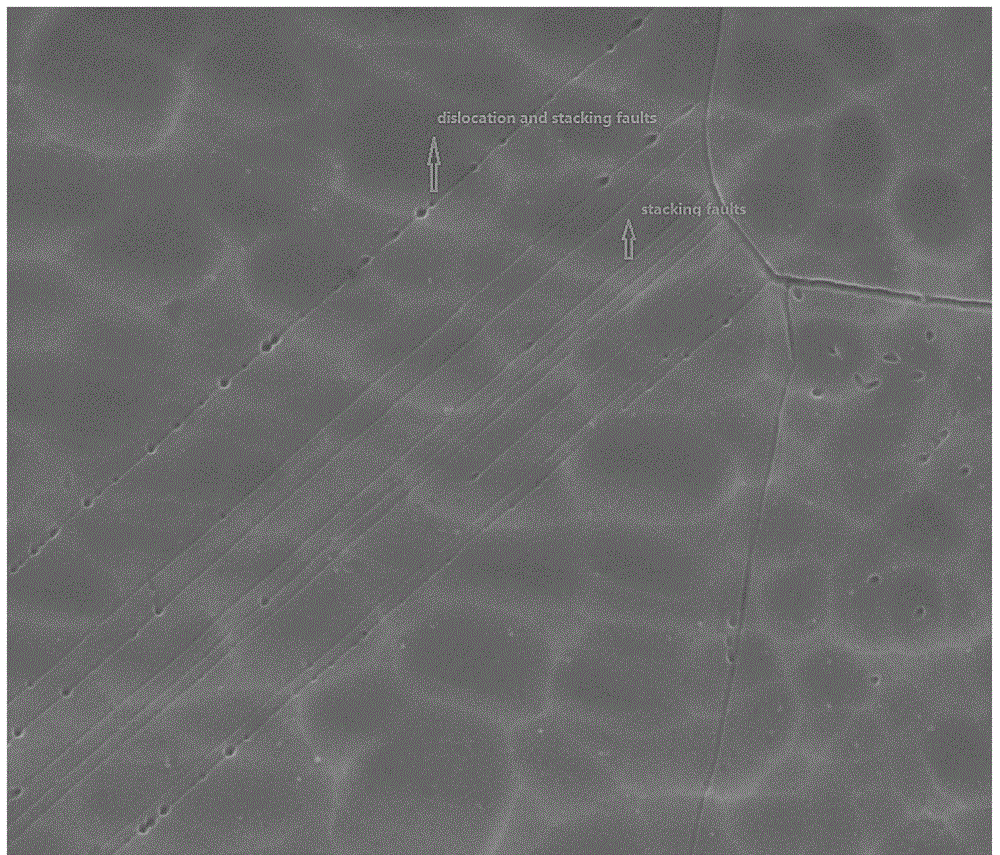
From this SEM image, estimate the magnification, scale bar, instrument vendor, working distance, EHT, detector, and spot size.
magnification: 5 K X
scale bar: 20000 nm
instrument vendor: FEI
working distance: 10 mm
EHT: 20 kV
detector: ETD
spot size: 3